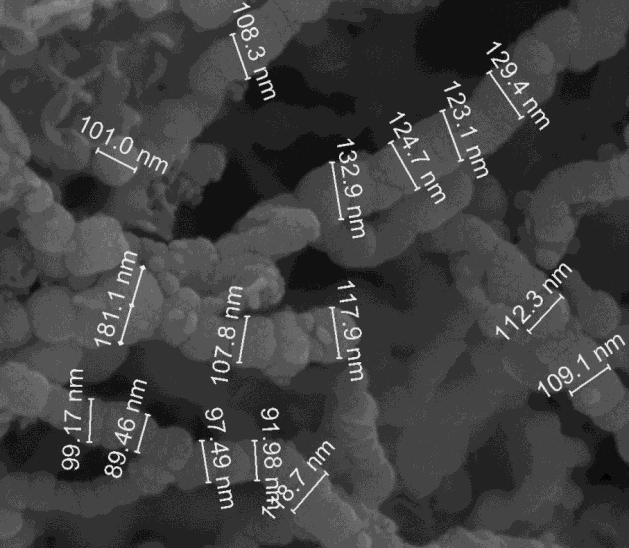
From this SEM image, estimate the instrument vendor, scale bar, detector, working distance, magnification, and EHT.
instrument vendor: FEI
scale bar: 400 nm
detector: ETD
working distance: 9.6 mm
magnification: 200 K X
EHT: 15.5 kV